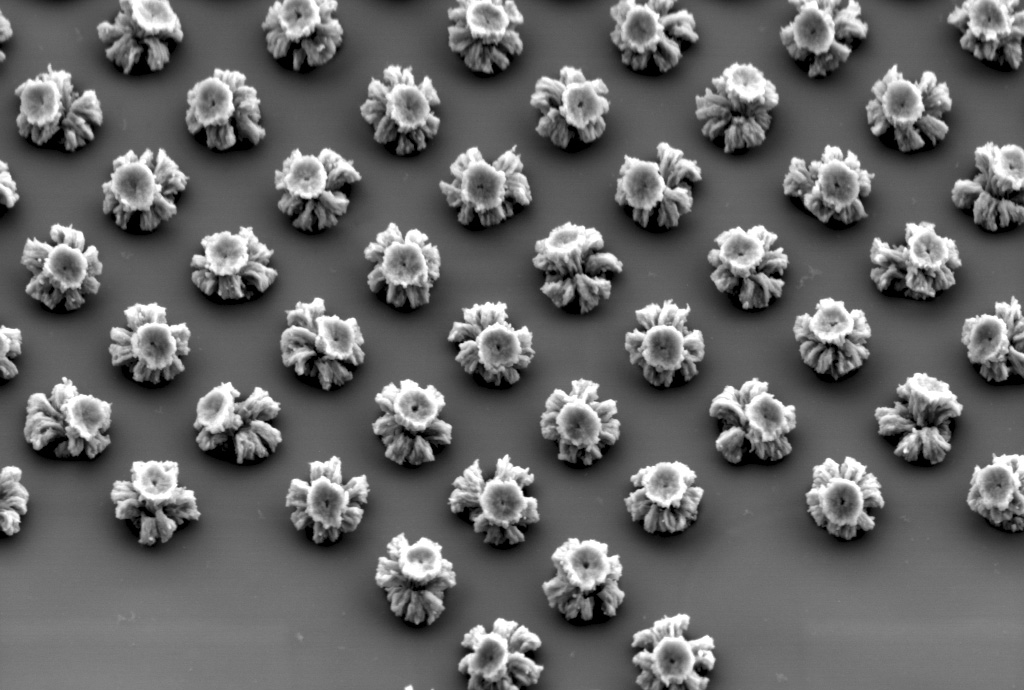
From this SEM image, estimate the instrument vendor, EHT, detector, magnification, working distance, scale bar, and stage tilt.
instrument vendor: Zeiss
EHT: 3 kV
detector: HE-SE2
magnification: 15.74 K X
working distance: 4.8 mm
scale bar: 1000 nm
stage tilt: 32.2°